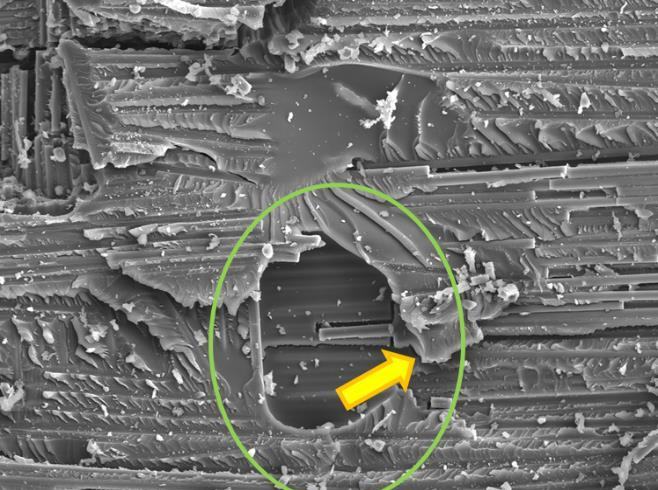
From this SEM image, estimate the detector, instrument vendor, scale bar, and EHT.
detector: SEI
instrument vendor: JEOL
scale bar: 50000 nm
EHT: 20 kV